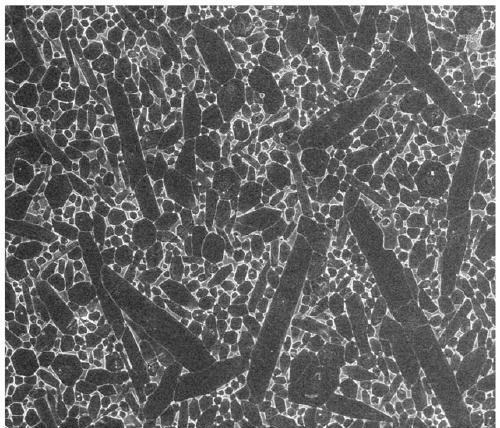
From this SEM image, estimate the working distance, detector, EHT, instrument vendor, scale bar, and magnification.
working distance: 3.8 mm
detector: TLD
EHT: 10 kV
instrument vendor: FEI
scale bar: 4000 nm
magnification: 20 K X